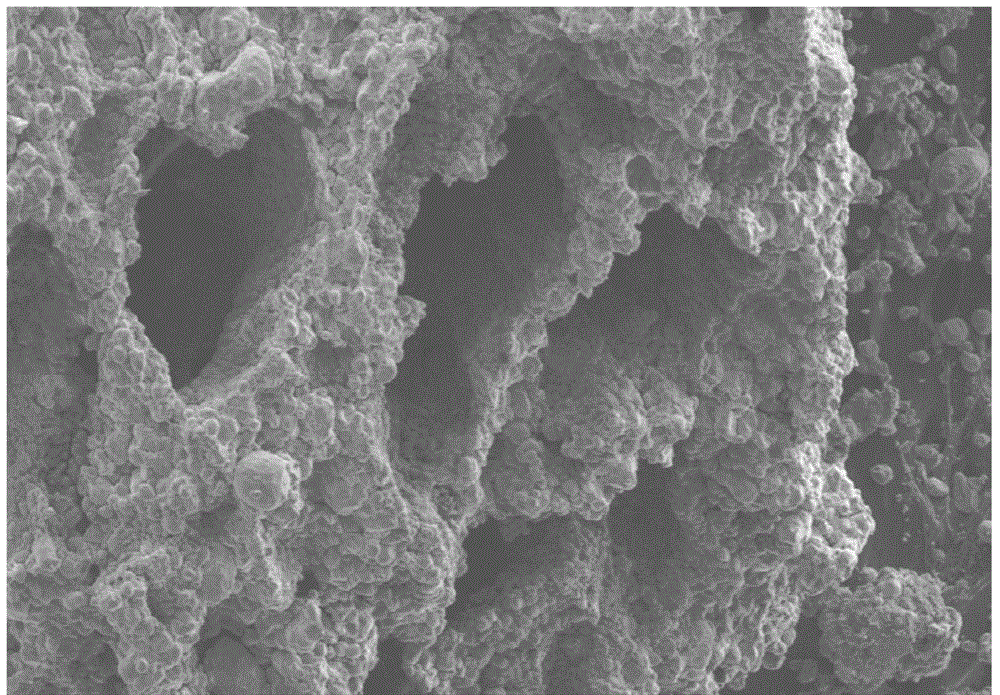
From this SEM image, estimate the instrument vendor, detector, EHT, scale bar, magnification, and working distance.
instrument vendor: Hitachi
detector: SE(M)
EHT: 15 kV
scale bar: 1000000 nm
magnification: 0.05 K X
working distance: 18.4 mm